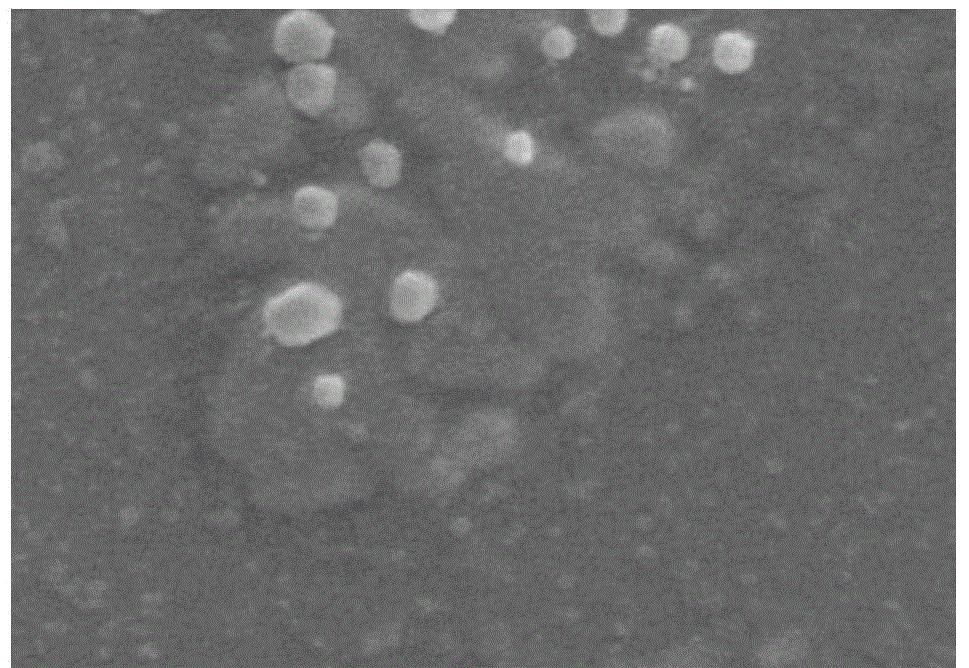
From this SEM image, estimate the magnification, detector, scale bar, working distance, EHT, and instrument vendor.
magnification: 15 K X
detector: SE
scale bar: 3000 nm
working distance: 7.2 mm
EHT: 15 kV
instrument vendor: Hitachi